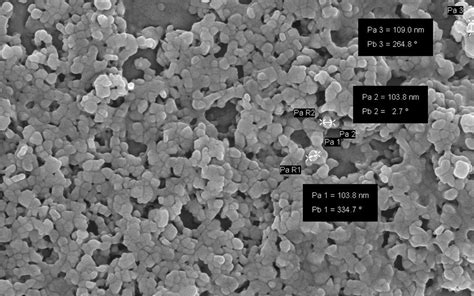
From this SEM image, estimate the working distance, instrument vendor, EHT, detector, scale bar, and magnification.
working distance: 3.9 mm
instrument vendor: Zeiss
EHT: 5 kV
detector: InLens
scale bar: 200 nm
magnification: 55 K X